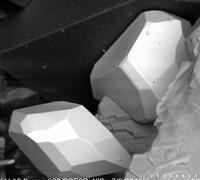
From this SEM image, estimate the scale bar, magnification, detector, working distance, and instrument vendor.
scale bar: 50000 nm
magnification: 0.6 K X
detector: BSE3D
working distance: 16.3 mm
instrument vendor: Hitachi